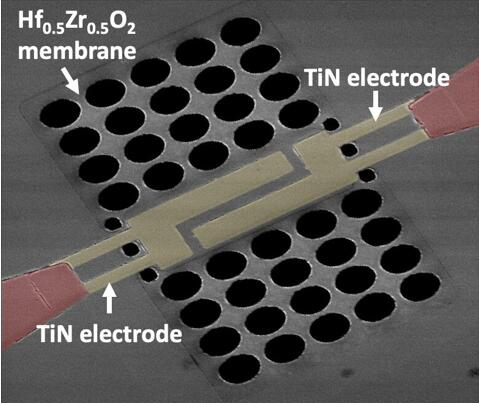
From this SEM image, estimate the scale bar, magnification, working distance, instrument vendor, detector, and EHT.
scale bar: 50000 nm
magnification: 1.2 K X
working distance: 15.6 mm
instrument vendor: FEI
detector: ETD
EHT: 1 kV